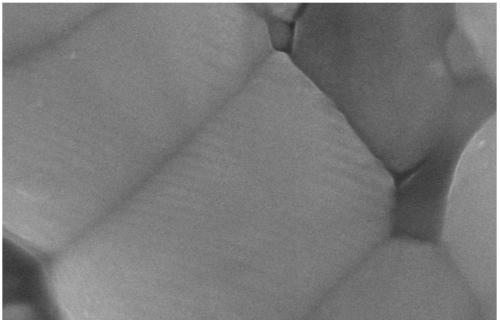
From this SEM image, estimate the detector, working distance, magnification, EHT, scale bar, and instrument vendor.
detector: SE(U)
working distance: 5.2 mm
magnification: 80 K X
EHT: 15 kV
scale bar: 500 nm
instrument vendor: Hitachi